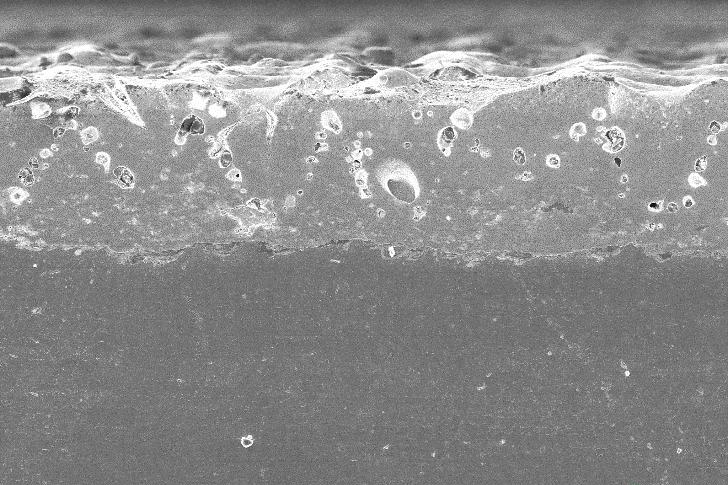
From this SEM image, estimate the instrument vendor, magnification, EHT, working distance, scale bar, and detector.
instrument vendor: Zeiss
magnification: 0.3 K X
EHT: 20 kV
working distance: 34 mm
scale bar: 100000 nm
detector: SE1